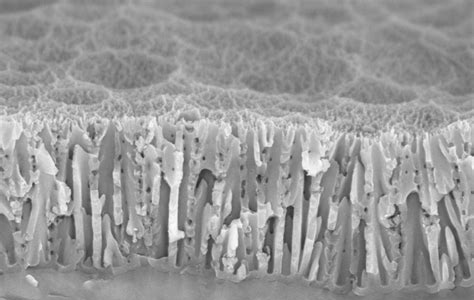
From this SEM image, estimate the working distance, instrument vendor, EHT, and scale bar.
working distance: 3.4 mm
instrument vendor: Zeiss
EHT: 5 kV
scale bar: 200 nm